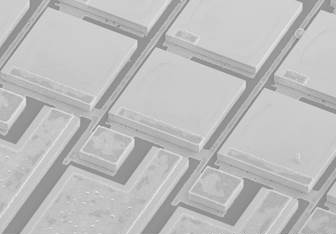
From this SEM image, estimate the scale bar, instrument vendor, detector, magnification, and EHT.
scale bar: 300000 nm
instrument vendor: Hitachi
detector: SE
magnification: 0.15 K X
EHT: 5 kV